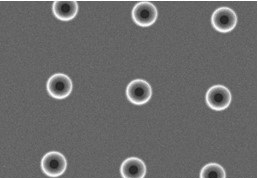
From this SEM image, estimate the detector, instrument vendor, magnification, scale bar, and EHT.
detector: SE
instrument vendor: Hitachi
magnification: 0.4 K X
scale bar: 100000 nm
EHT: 15 kV